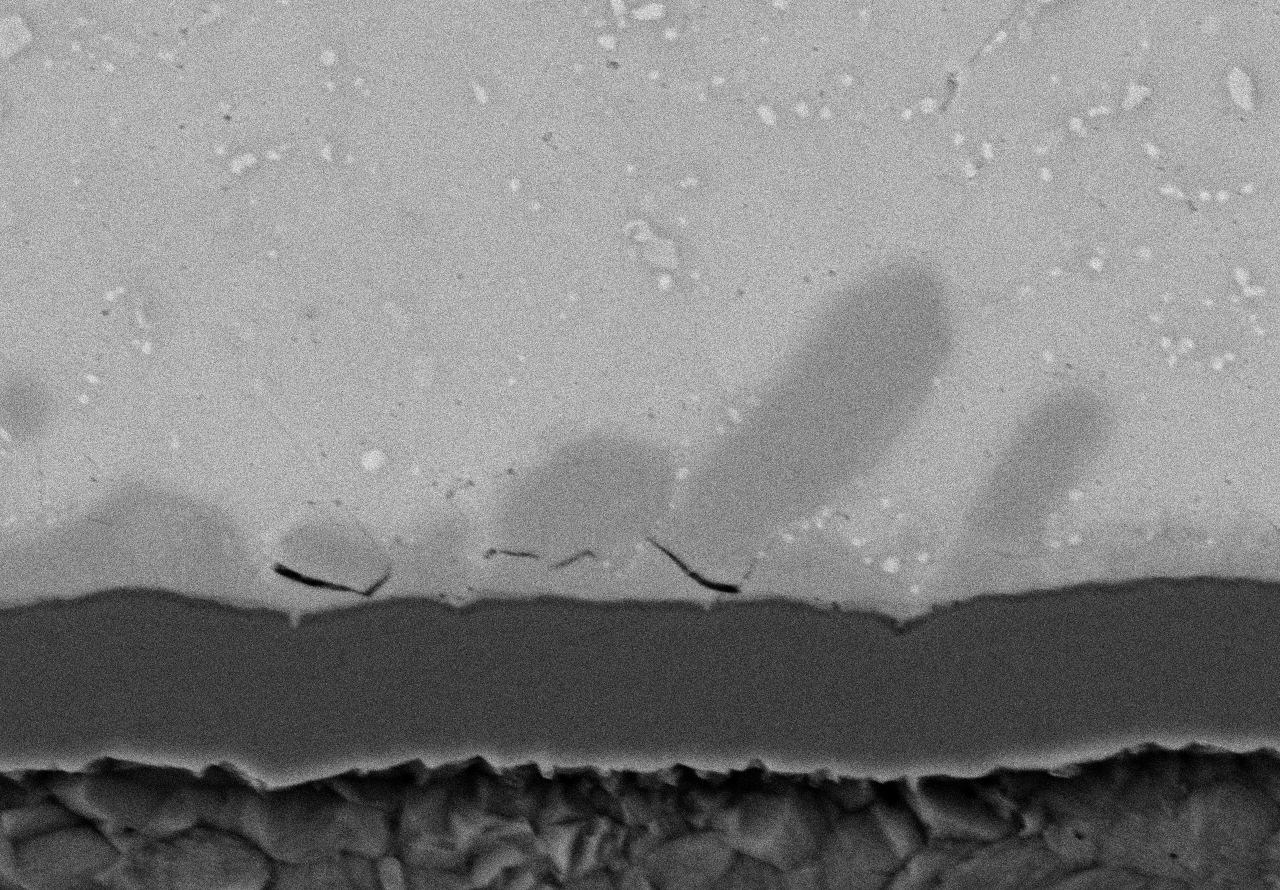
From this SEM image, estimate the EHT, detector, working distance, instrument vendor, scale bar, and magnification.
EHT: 15 kV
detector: BSE3D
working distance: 6.9 mm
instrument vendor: Hitachi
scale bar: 10000 nm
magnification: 5 K X